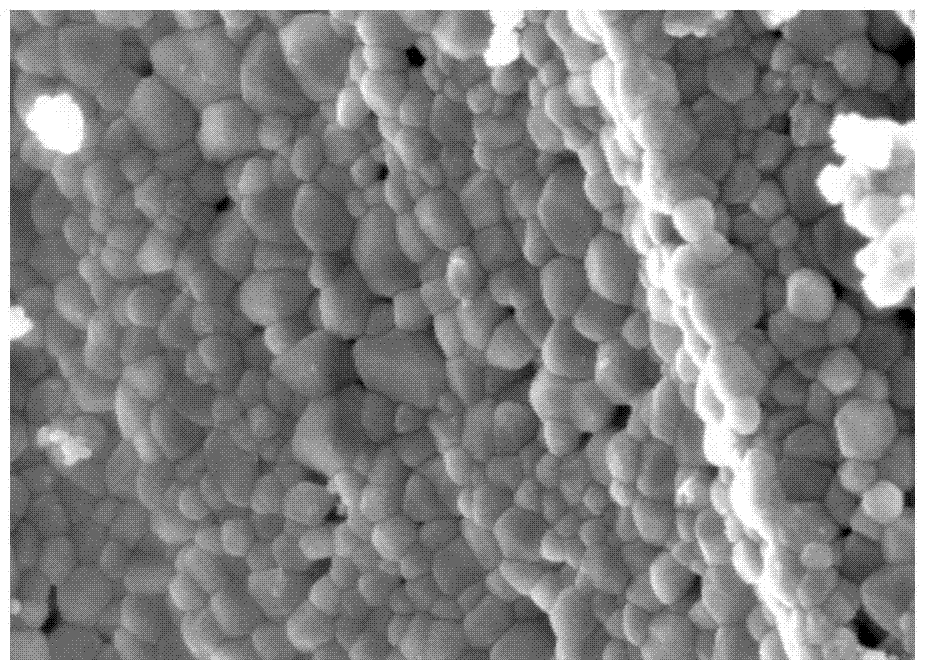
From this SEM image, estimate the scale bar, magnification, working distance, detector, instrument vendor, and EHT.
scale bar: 1000 nm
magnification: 50 K X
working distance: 7.7 mm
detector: SE(M)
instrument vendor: Hitachi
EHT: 5 kV